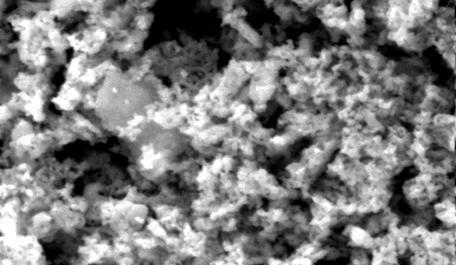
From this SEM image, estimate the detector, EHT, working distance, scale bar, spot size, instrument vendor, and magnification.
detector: SE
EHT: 20 kV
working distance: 6.6 mm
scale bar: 2000 nm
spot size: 4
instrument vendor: FEI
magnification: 10 K X